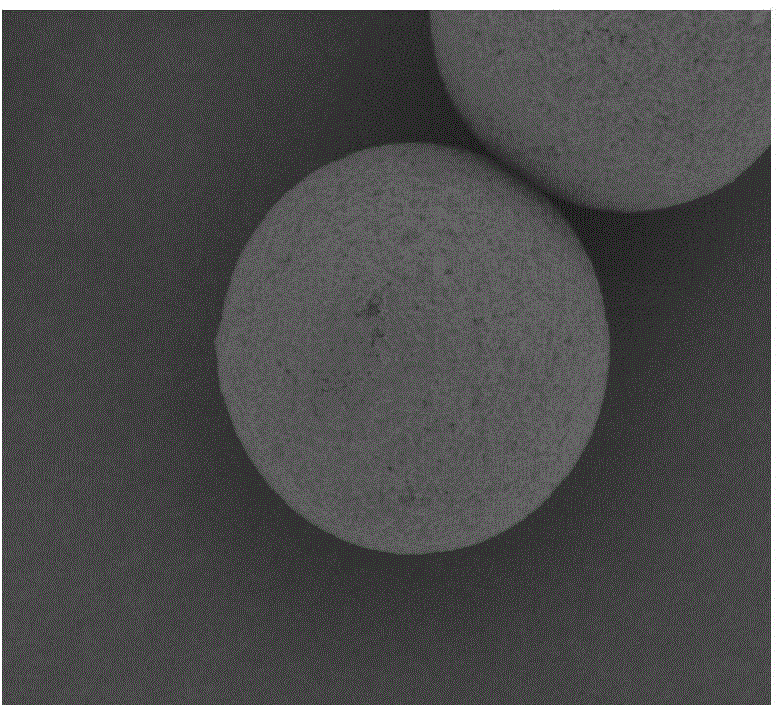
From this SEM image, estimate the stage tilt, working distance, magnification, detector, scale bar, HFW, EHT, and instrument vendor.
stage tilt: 0°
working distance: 11 mm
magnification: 5 K X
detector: BSED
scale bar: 20000 nm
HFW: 59.7 µm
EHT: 15 kV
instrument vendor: FEI